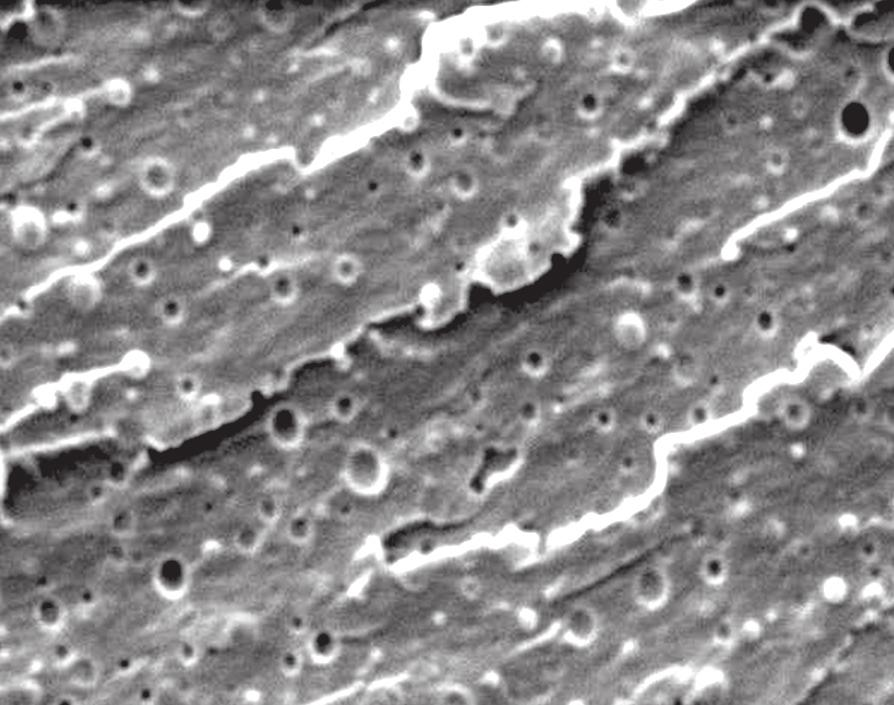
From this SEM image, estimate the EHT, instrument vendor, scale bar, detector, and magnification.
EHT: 5 kV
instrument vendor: JEOL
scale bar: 1000 nm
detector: SEI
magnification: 10 K X